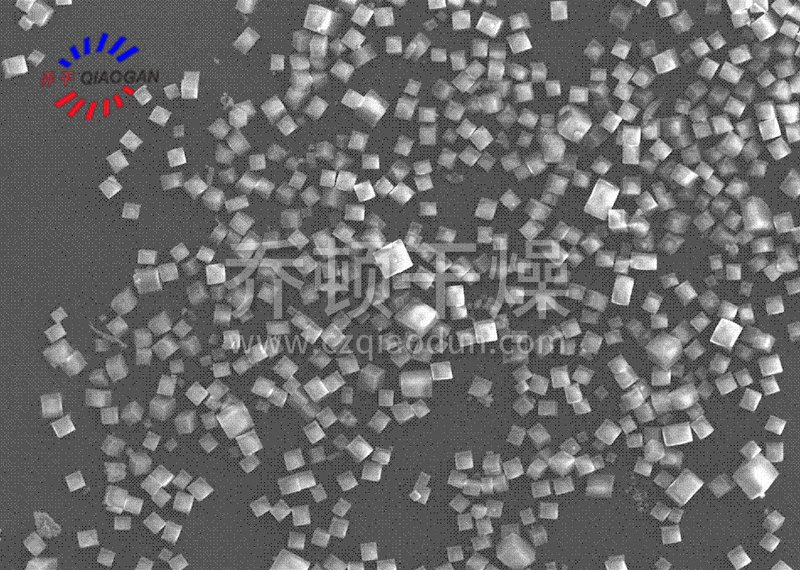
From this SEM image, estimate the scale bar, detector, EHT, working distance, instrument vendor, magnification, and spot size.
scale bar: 50000 nm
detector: SEI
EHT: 10 kV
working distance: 17 mm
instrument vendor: JEOL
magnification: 0.5 K X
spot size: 8